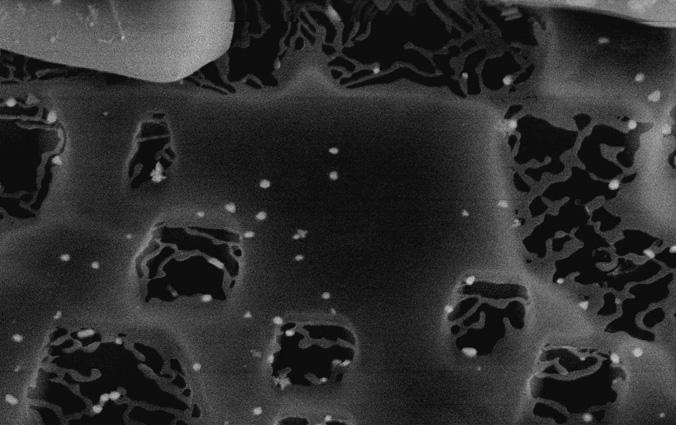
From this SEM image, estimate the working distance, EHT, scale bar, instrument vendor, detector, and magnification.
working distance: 15 mm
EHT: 10 kV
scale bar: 3000 nm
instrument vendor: JEOL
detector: SE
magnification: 20 K X